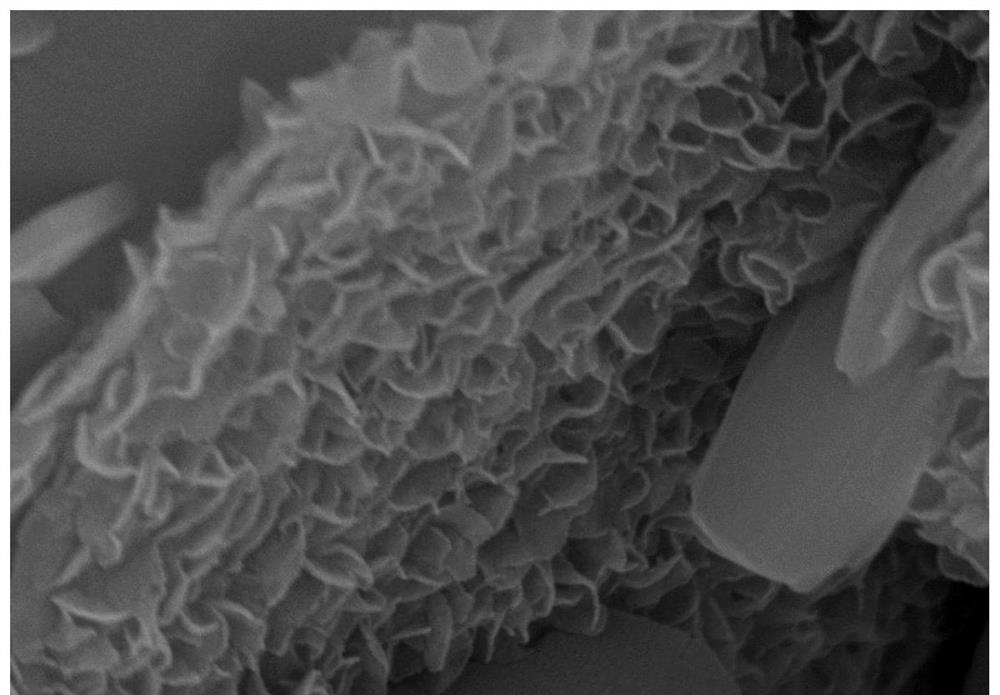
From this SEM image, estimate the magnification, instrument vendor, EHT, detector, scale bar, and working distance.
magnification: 80 K X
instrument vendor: Hitachi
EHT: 10 kV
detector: SE(U)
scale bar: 500 nm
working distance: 8.5 mm